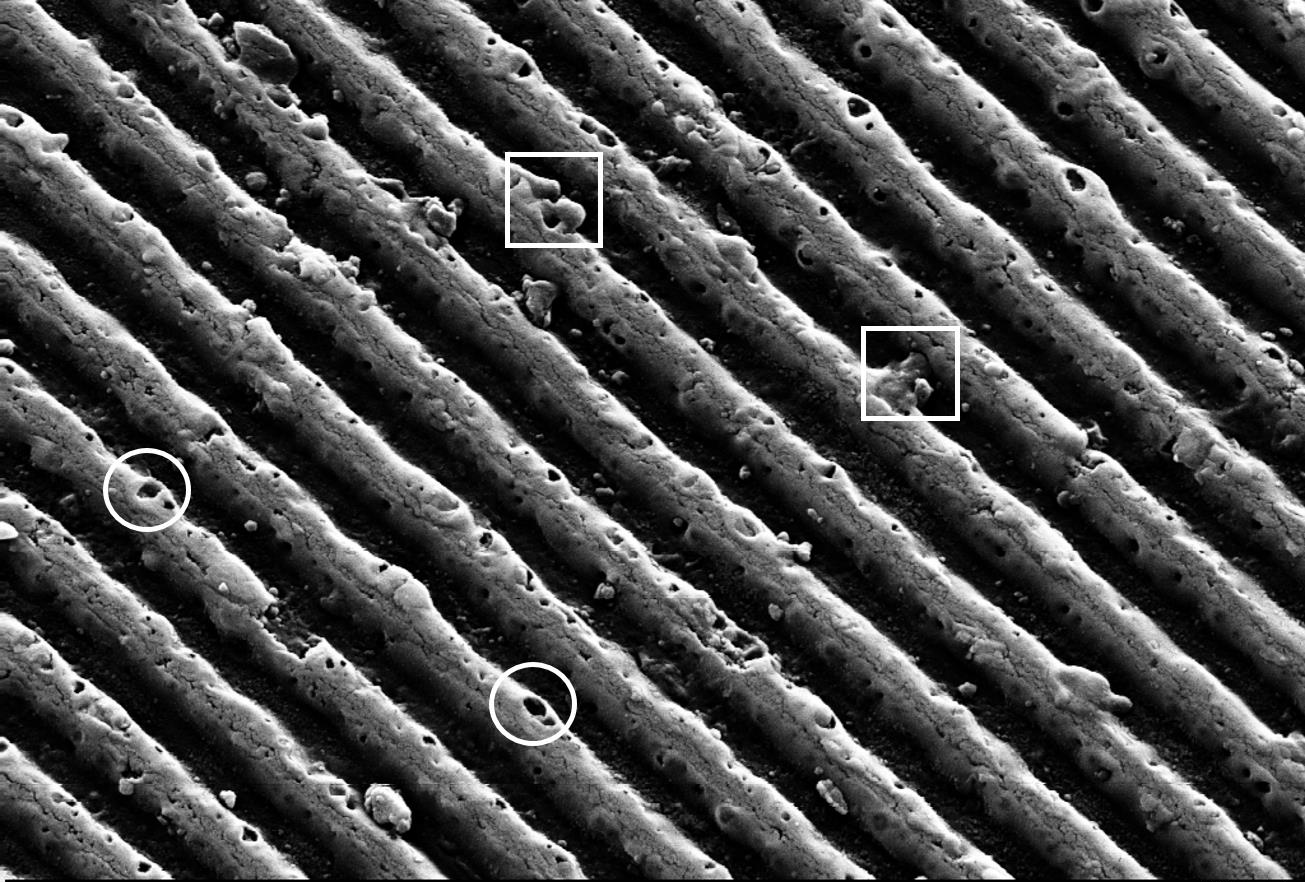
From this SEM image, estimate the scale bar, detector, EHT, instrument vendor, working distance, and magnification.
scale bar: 1000 nm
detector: SE2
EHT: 18 kV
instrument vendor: Zeiss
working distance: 5.9 mm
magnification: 20 K X